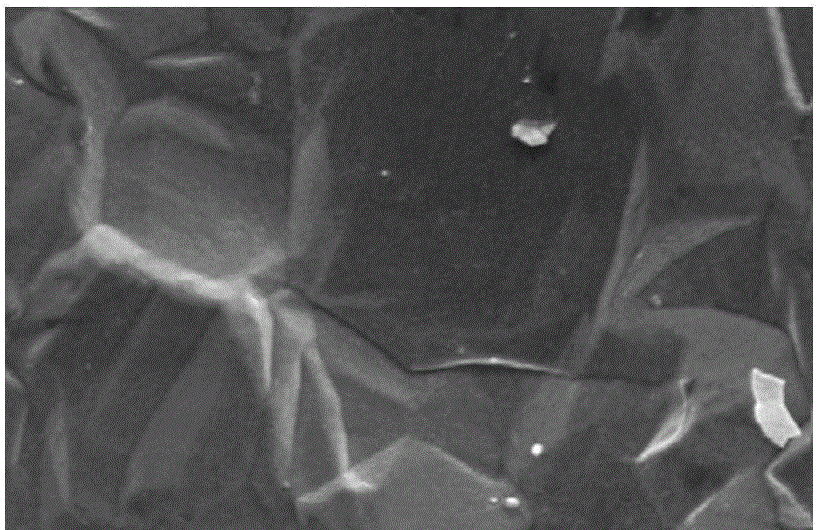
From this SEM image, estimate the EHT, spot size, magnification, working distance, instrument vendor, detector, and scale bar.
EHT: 5 kV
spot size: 3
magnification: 10 K X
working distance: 7.5 mm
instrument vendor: FEI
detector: SE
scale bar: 5000 nm